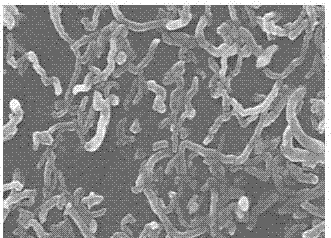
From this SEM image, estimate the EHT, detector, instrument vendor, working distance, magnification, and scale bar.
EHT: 5 kV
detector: SEI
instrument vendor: JEOL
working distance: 3.4 mm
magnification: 50 K X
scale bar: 100 nm